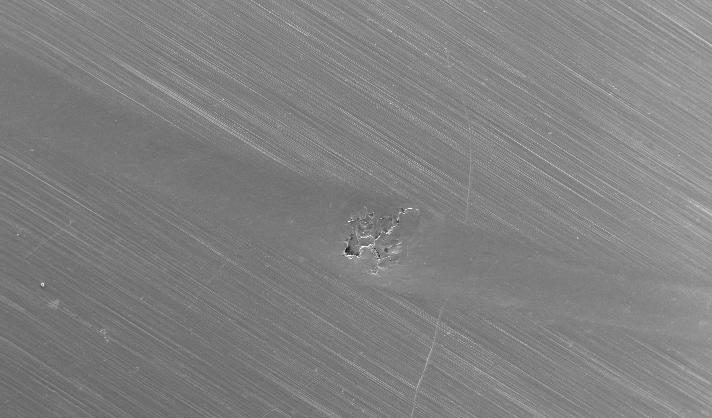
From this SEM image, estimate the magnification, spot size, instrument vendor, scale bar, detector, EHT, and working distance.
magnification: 0.025 K X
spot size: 4.2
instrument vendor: FEI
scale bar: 1000000 nm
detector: SE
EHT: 20 kV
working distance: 12.2 mm